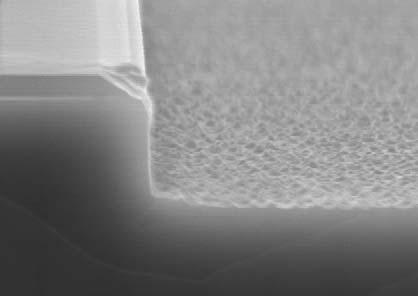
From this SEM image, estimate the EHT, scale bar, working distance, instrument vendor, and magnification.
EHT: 15 kV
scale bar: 1000 nm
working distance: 11.5 mm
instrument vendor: Hitachi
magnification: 30 K X